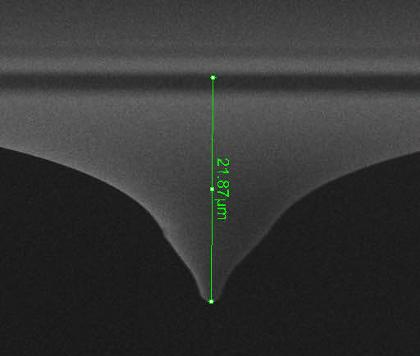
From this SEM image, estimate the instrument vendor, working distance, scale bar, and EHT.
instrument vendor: FEI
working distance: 15.2 mm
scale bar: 10000 nm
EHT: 10 kV